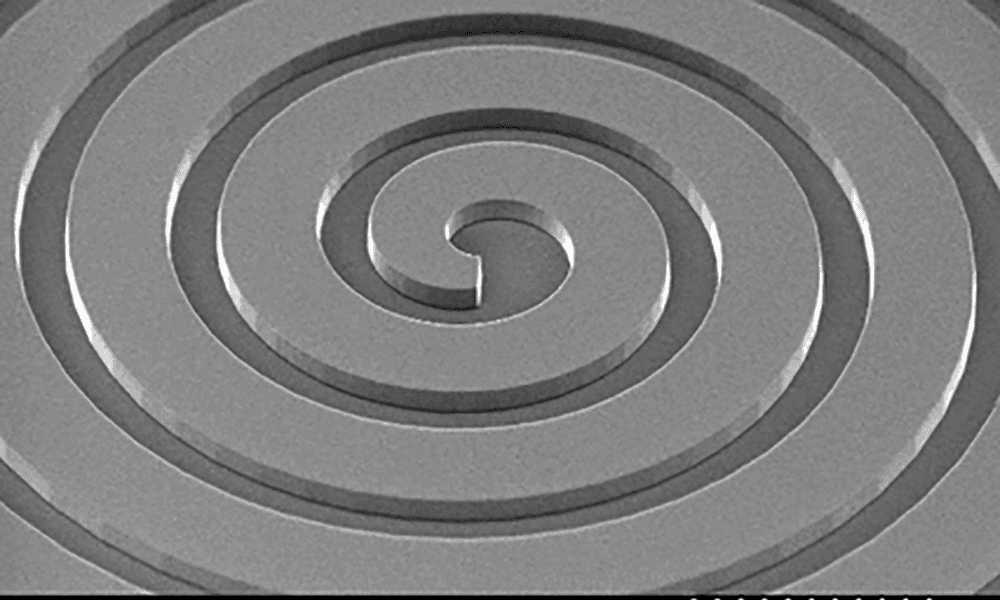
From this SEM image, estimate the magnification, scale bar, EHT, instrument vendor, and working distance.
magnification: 0.06 K X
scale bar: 500000 nm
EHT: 15 kV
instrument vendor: JEOL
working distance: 10.1 mm